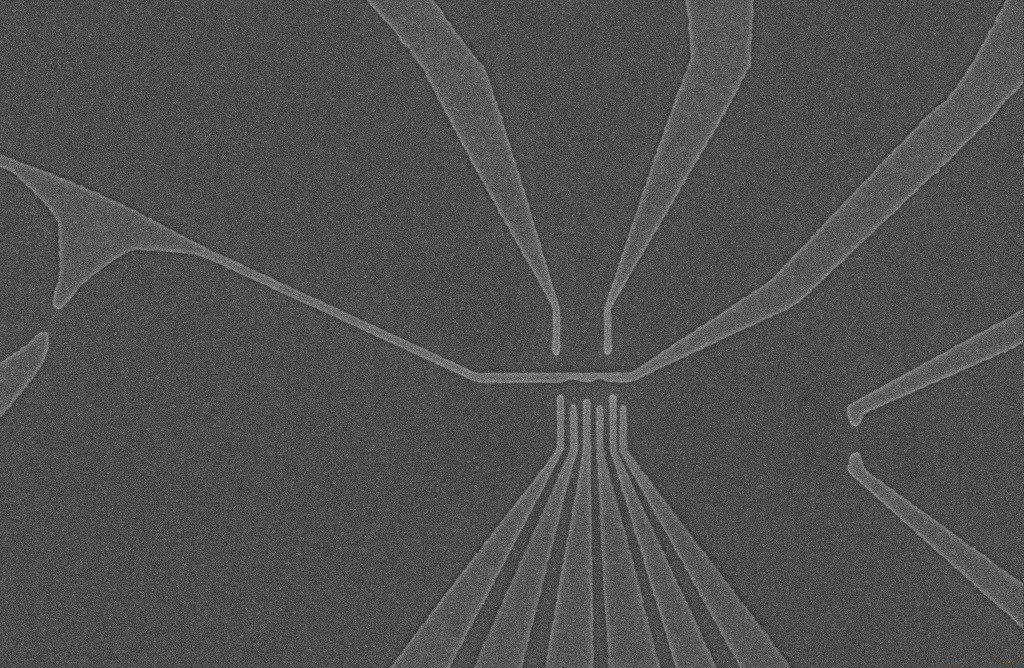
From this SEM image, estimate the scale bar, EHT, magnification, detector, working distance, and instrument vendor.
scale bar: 1000 nm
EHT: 5 kV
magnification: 11.62 K X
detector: InLens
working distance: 8.4 mm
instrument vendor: Zeiss